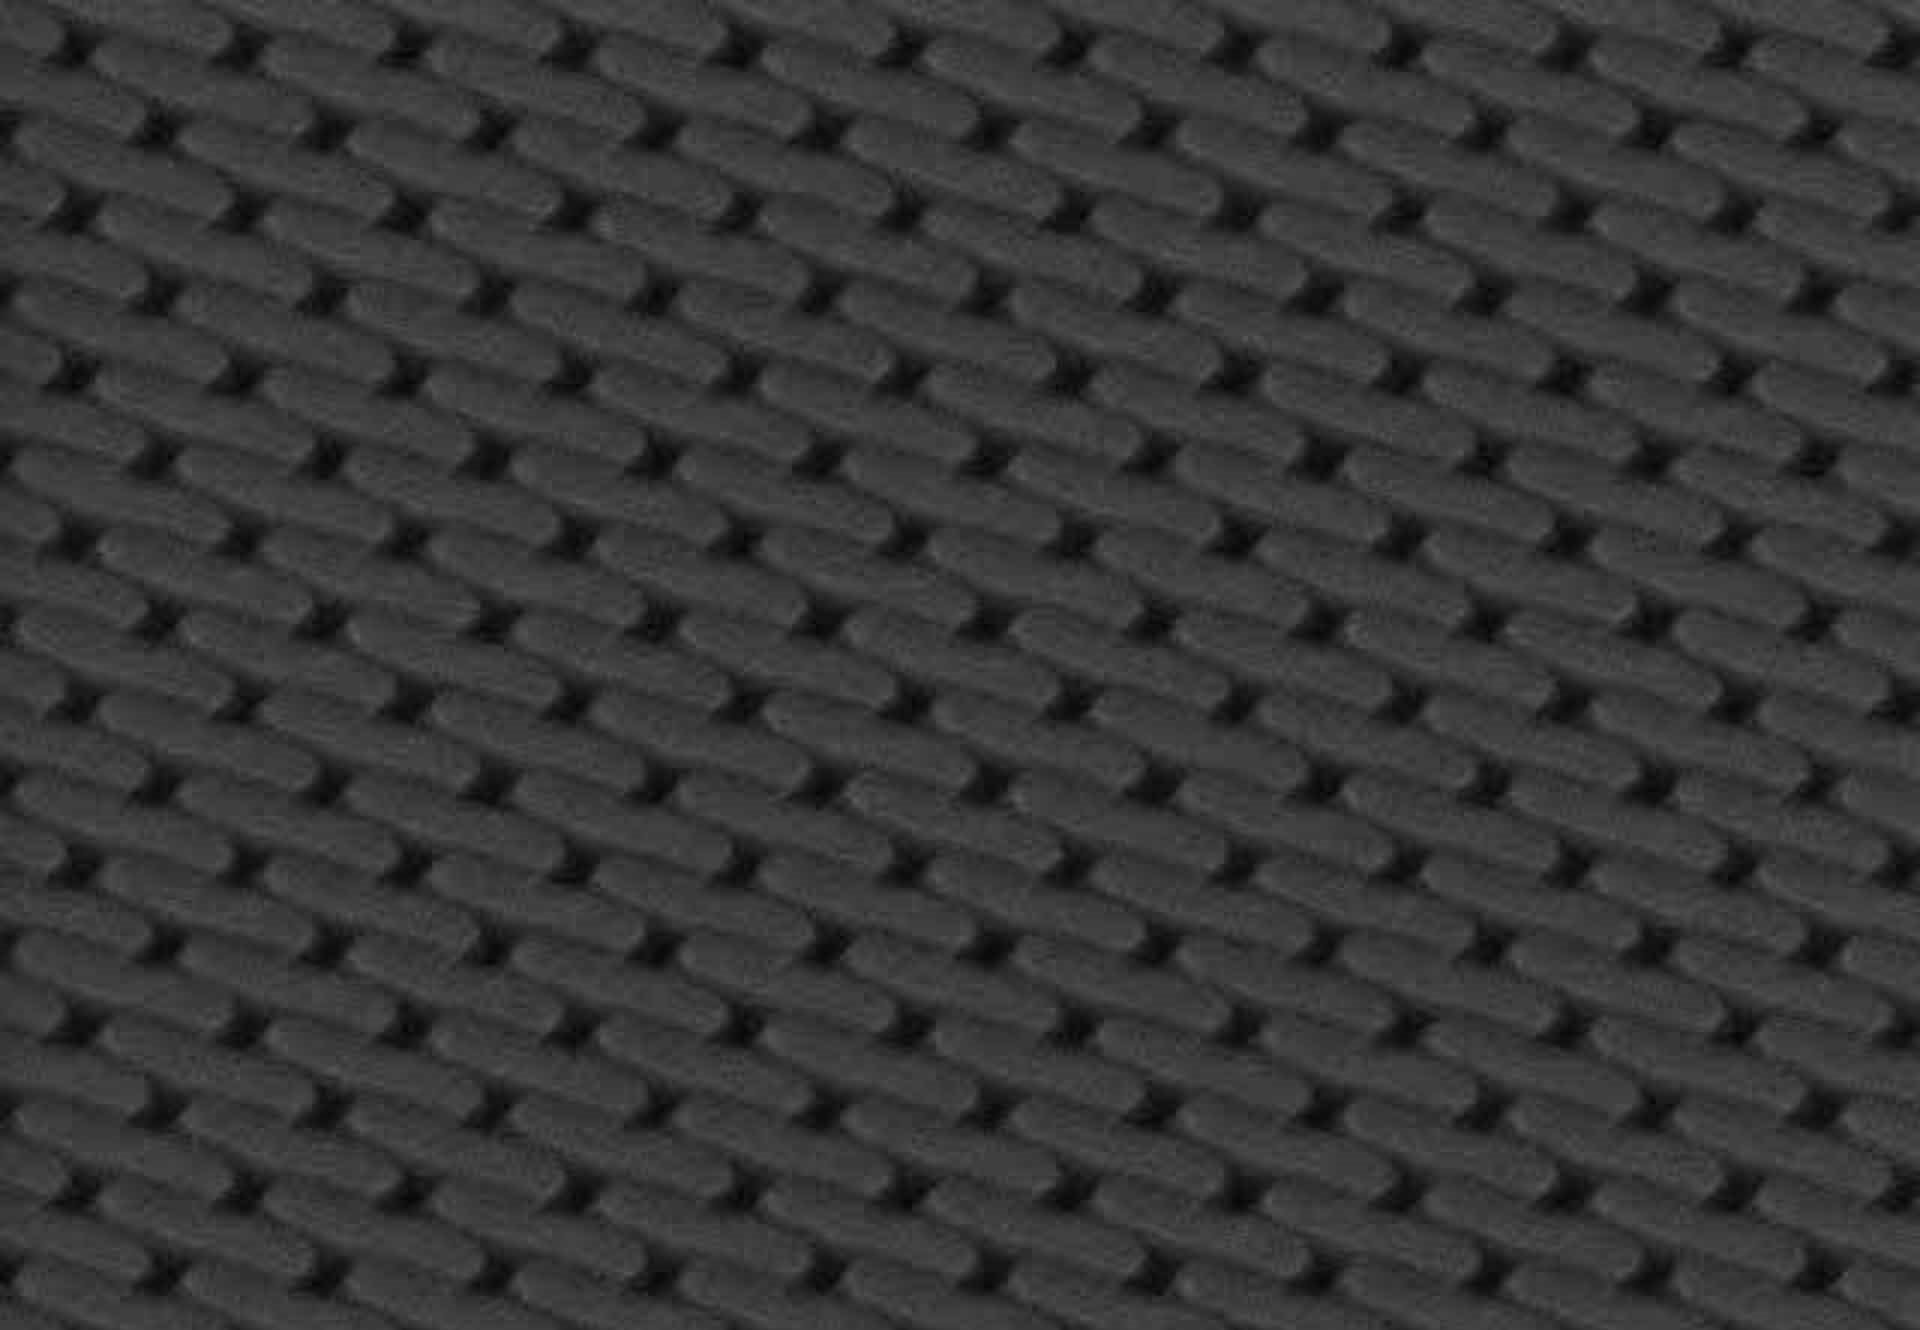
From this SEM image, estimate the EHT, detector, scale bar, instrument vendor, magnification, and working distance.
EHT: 15 kV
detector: SE(M)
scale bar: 500 nm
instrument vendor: Hitachi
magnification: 70.6 K X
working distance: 18.7 mm